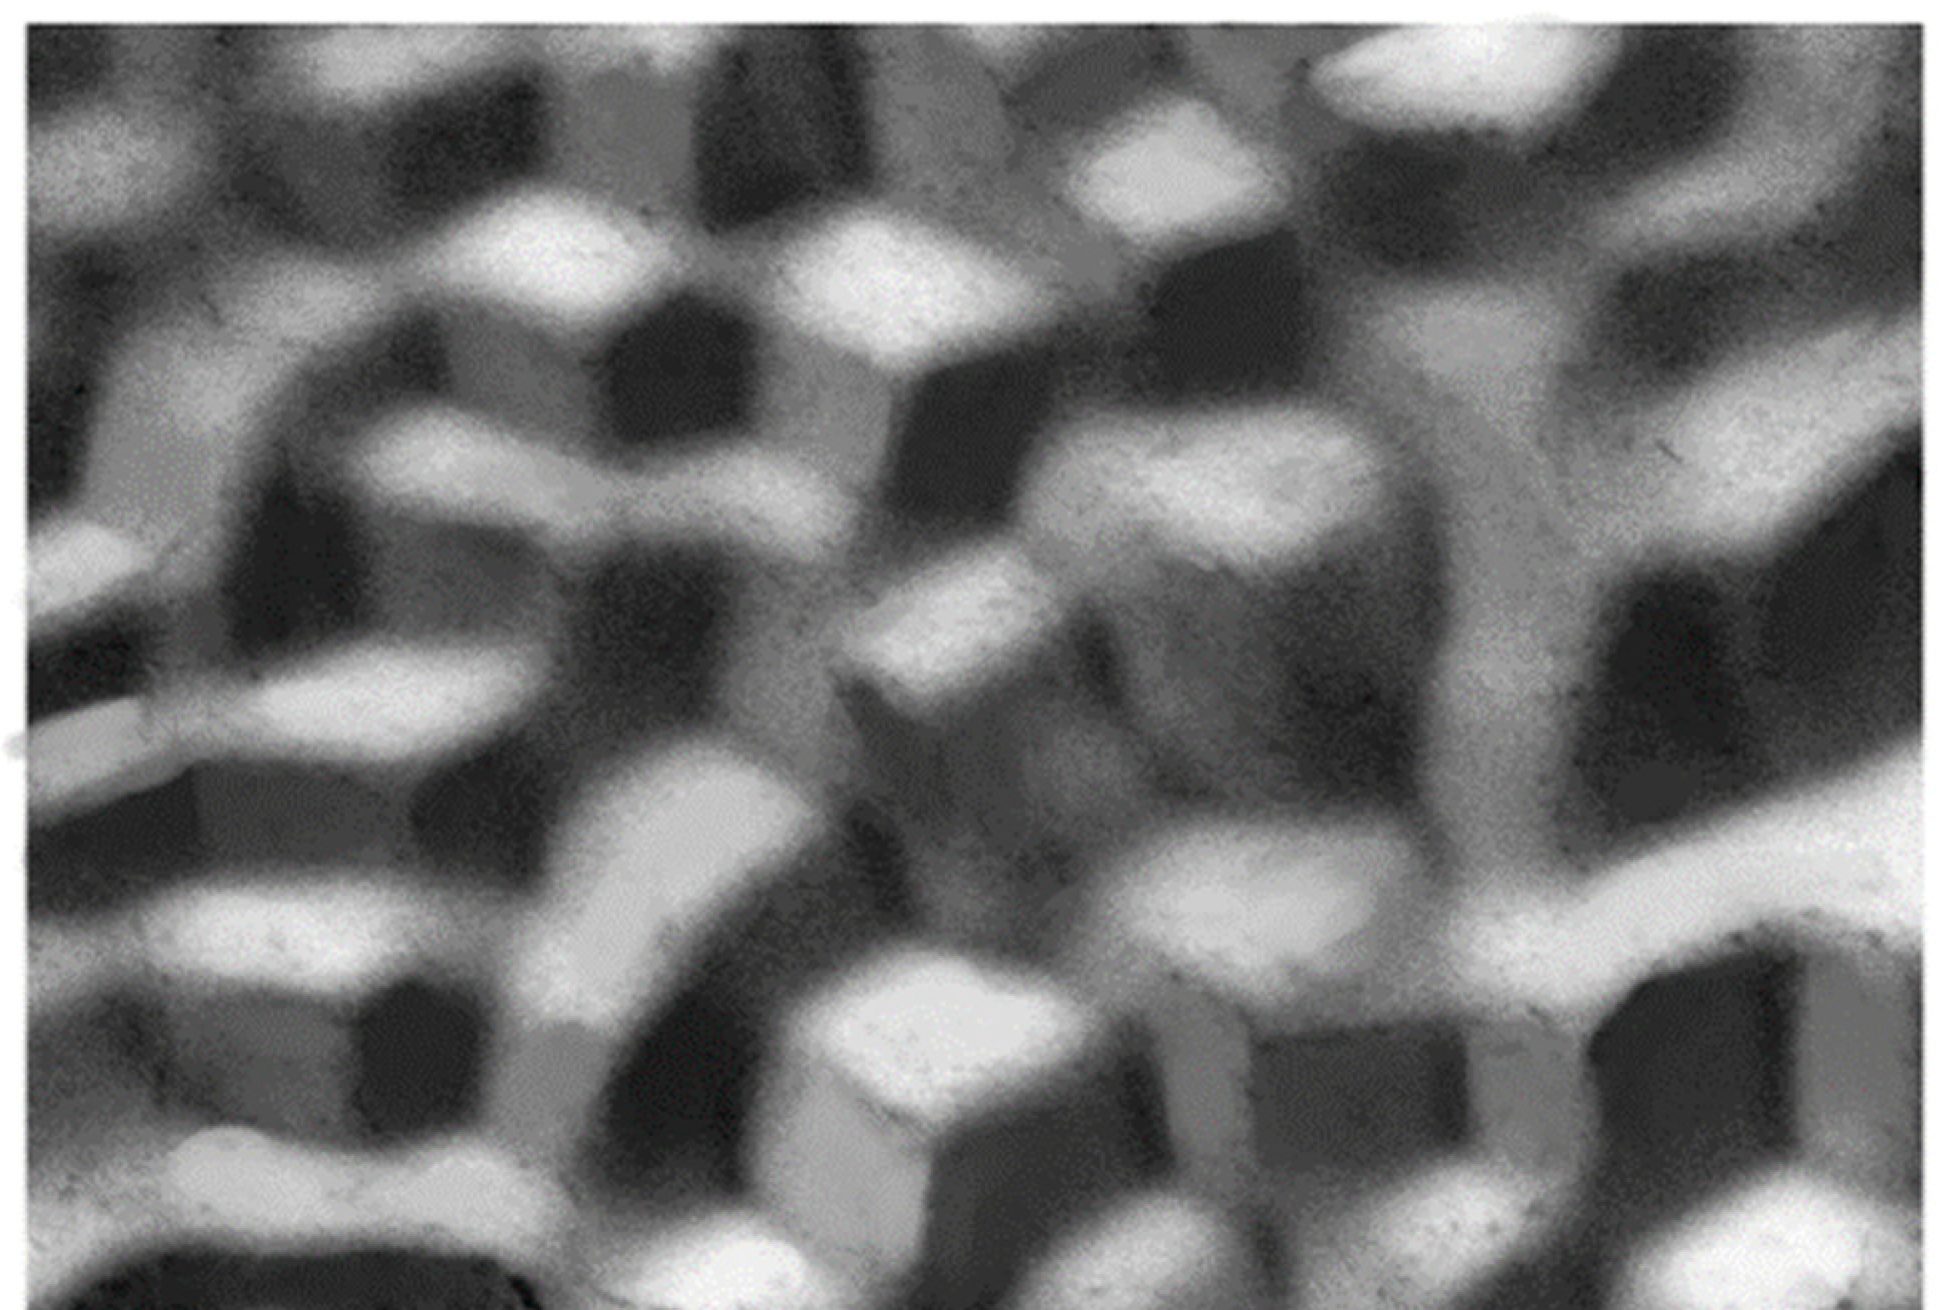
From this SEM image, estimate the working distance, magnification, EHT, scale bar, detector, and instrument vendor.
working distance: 11 mm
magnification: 2 K X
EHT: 15 kV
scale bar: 10000 nm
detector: SEI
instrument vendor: JEOL